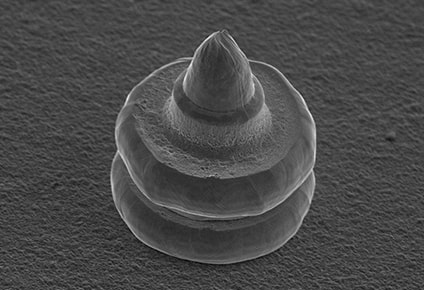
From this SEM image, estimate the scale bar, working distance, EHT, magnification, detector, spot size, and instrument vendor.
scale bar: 20000 nm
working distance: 11 mm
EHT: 15 kV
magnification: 0.85 K X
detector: SEI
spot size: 50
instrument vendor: JEOL